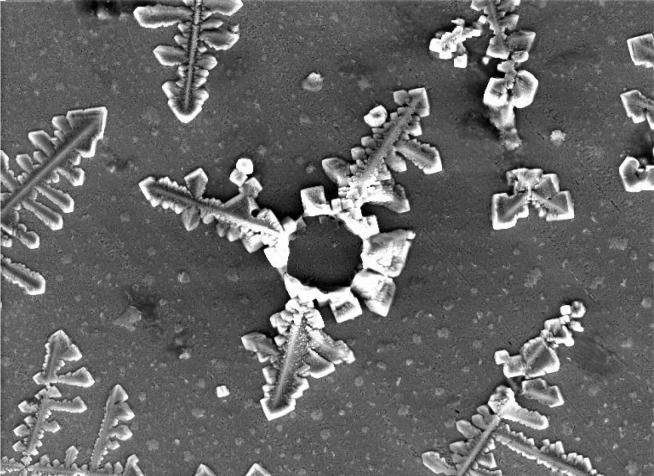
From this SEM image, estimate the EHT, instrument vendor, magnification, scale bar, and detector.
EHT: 30 kV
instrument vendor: other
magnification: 0.25 K X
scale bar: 200000 nm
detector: SE Detector